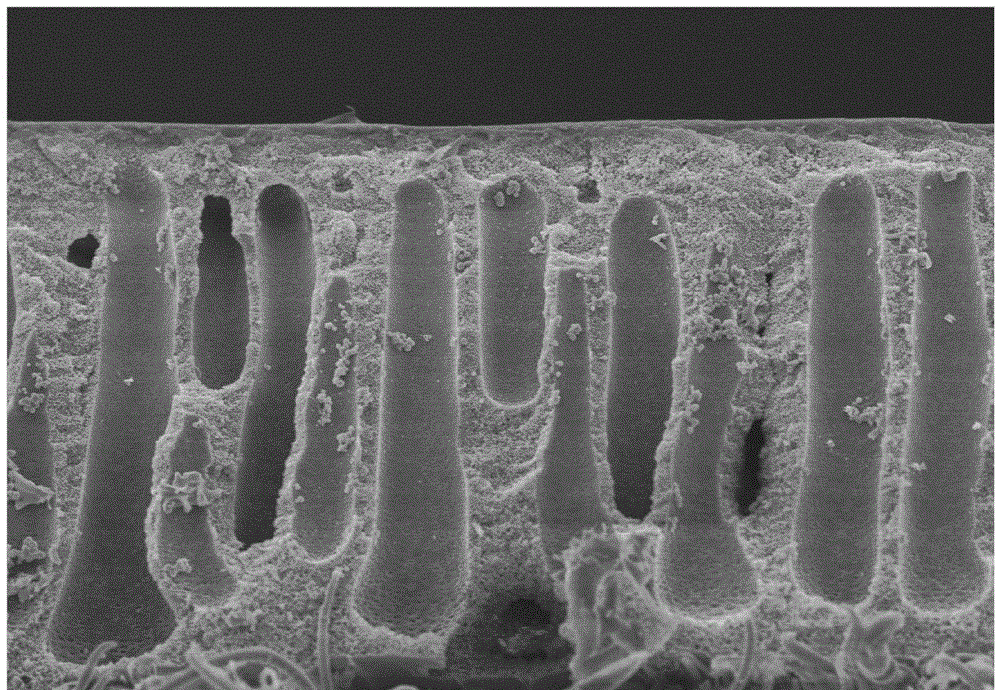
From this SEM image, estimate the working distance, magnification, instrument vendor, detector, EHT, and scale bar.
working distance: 8.8 mm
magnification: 1.5 K X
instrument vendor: Hitachi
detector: SE(M)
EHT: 5 kV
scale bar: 30000 nm